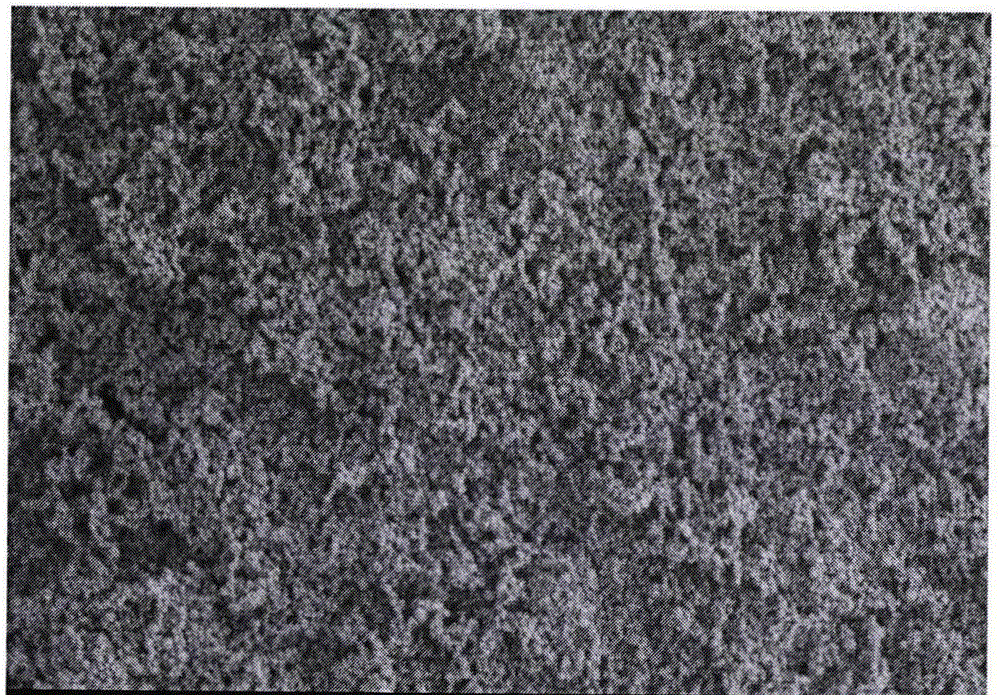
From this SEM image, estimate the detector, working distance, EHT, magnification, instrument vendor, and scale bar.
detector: SE(M,LA100)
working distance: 7.8 mm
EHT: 1 kV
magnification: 20 K X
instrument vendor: Hitachi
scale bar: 2000 nm